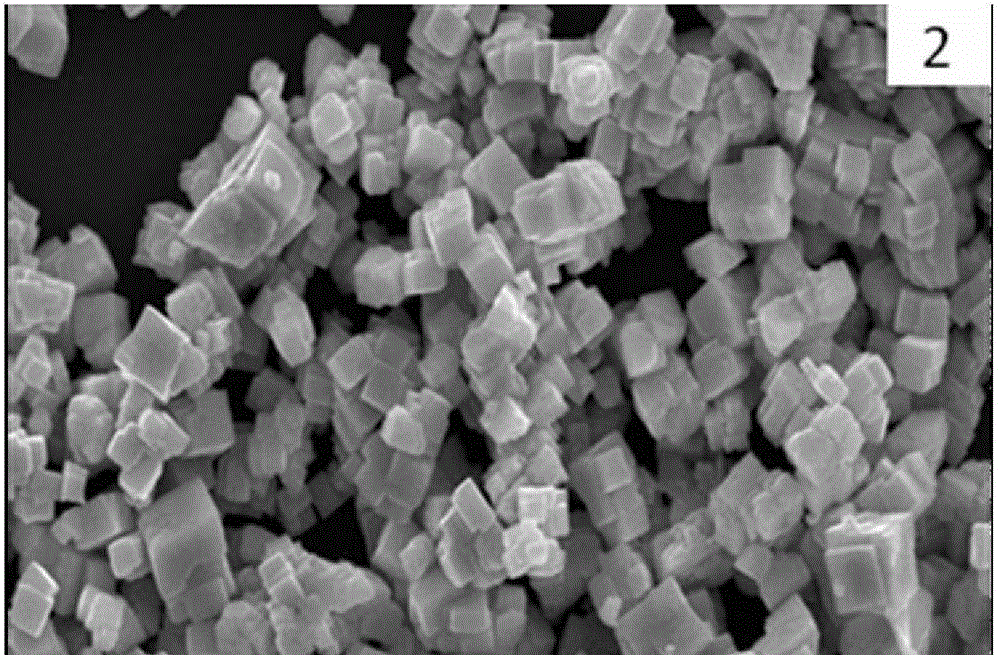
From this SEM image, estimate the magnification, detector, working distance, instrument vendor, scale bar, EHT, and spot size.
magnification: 5 K X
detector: SEI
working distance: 11 mm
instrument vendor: JEOL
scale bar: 5000 nm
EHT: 20 kV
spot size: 30